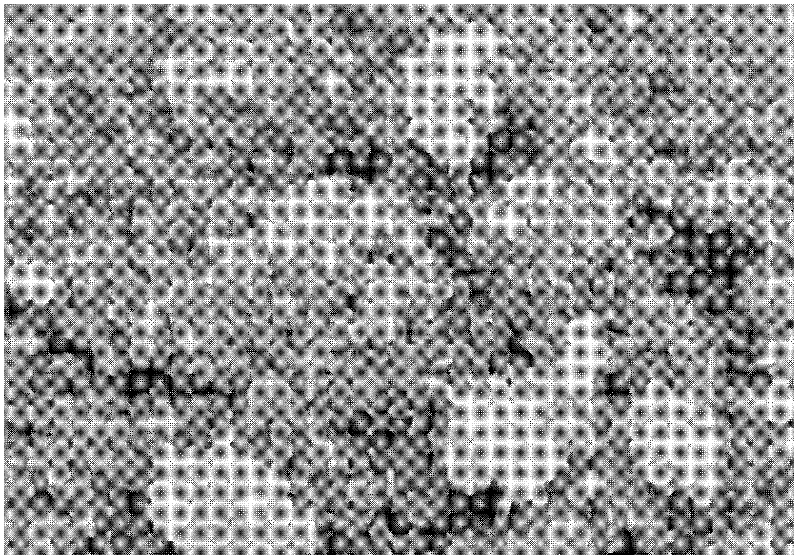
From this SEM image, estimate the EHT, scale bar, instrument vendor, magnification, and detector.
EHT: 5 kV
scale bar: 500 nm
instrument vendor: Hitachi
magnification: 80 K X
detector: SE(M)